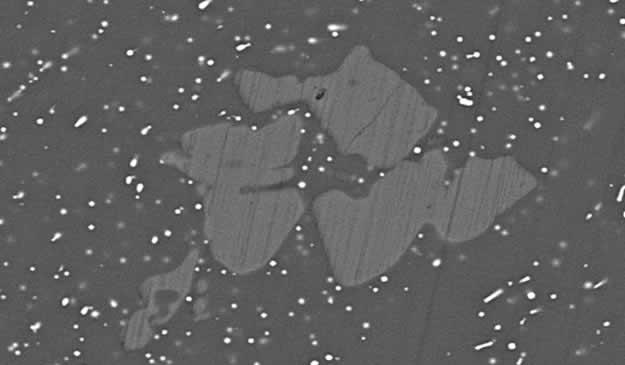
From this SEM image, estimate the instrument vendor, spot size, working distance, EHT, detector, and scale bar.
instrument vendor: FEI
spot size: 4.7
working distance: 10.1 mm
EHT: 20 kV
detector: BSE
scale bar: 10000 nm